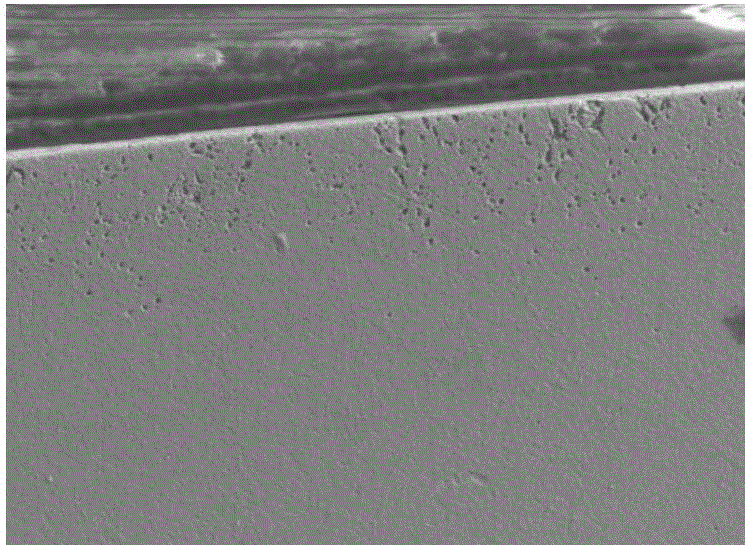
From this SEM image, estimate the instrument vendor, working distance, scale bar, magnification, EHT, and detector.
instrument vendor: JEOL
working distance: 10.7 mm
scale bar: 10000 nm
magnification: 0.5 K X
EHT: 15 kV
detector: SEI